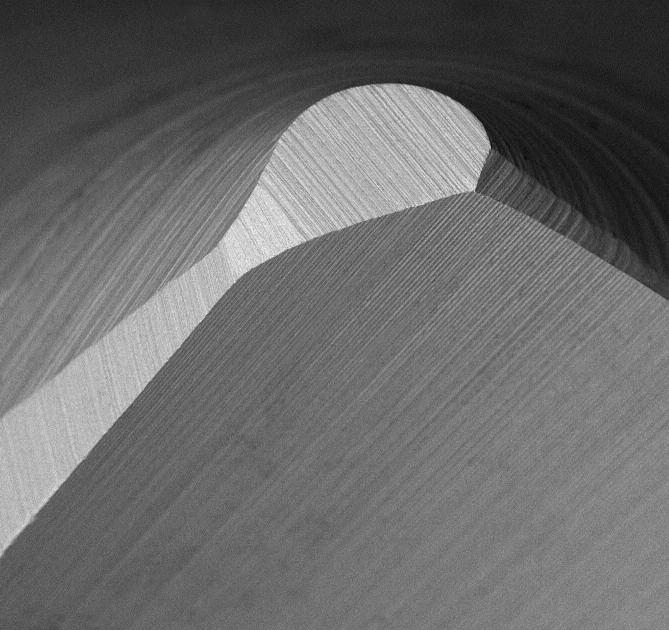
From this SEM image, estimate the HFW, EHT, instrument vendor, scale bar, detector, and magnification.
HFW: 268 µm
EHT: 15 kV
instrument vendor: other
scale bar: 80000 nm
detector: BSD Full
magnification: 1 K X